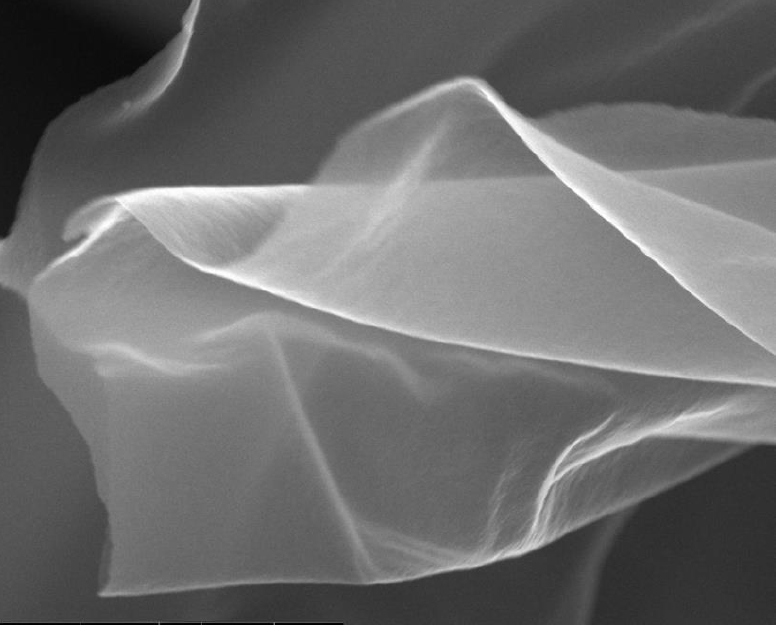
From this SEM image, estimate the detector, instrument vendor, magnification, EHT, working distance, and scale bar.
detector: TLD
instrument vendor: FEI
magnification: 24.976 K X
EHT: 10 kV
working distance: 4 mm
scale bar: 2000 nm